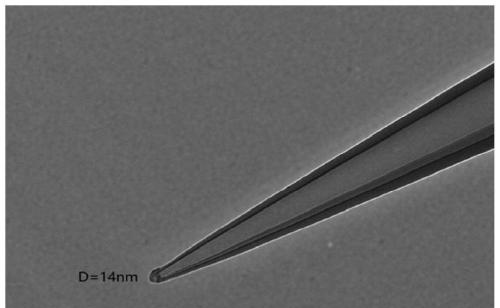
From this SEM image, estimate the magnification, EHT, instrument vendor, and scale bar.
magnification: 30 K X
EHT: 100 kV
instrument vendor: Hitachi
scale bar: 200 nm